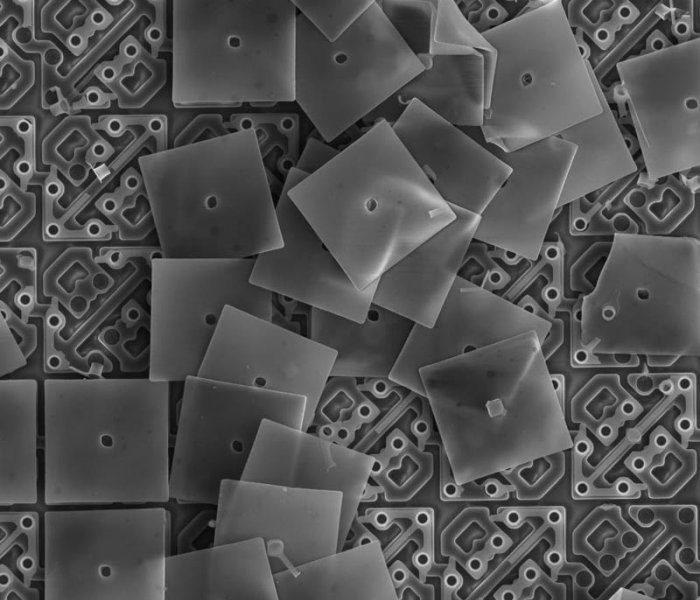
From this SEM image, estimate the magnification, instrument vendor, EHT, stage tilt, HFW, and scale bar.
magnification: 3.5 K X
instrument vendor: FEI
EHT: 10 kV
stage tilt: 0°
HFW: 73.1 µm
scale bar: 30000 nm